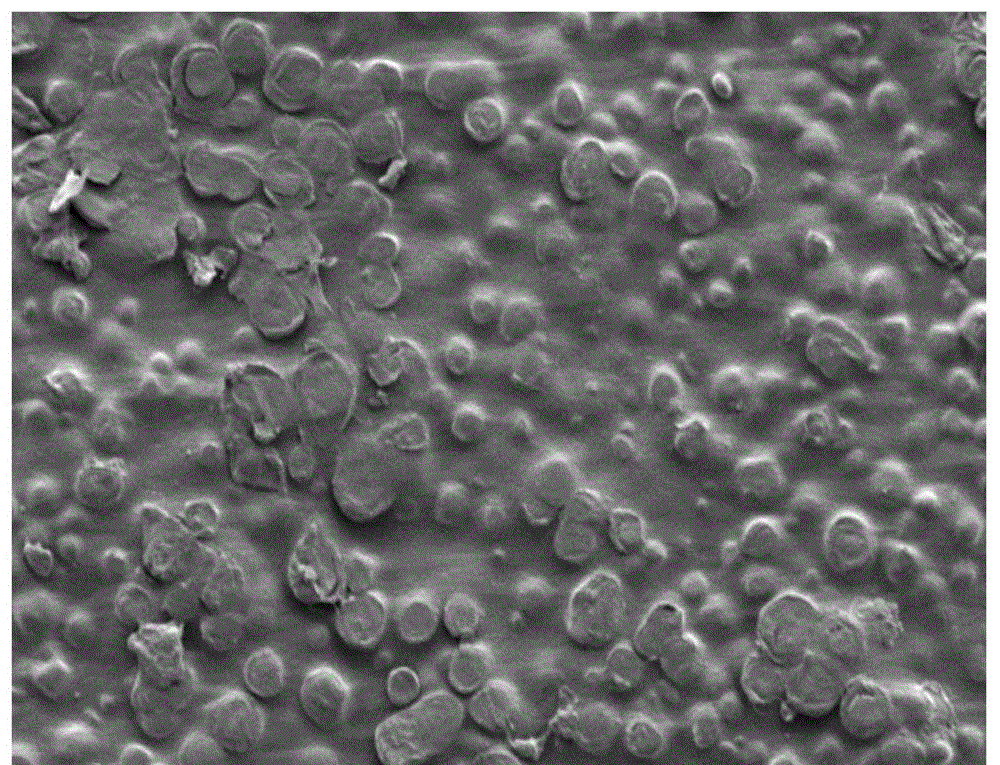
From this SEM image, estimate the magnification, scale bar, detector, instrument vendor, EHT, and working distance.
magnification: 0.35 K X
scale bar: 100000 nm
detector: LM(L)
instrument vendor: Hitachi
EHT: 3 kV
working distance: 12.3 mm